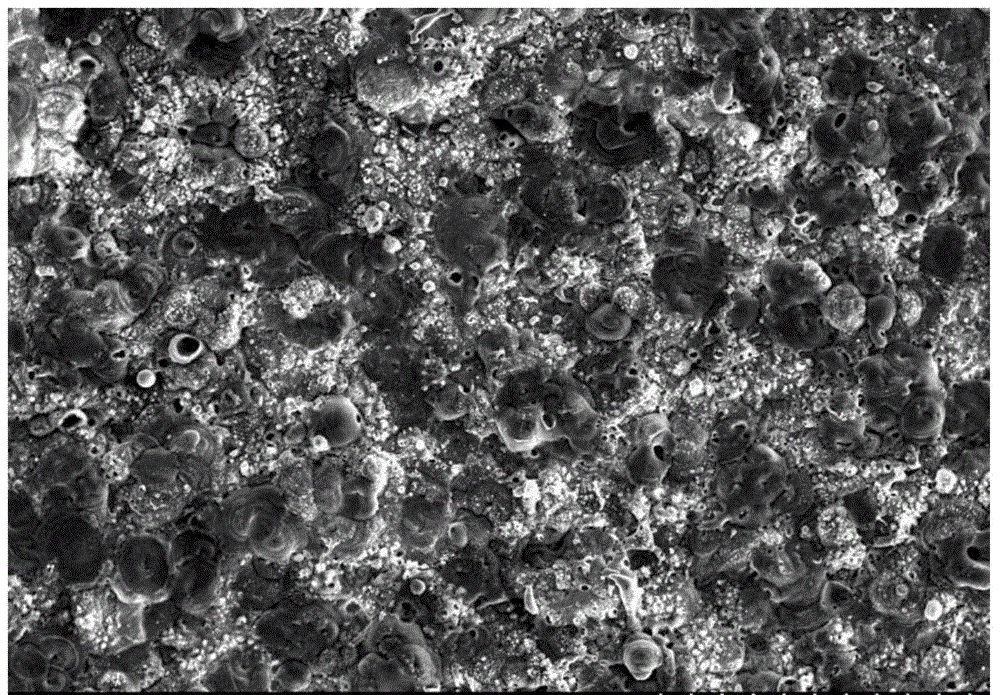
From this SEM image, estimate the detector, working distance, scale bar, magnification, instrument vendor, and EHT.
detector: SE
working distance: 9.3 mm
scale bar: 200000 nm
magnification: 0.2 K X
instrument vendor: Hitachi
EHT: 20 kV